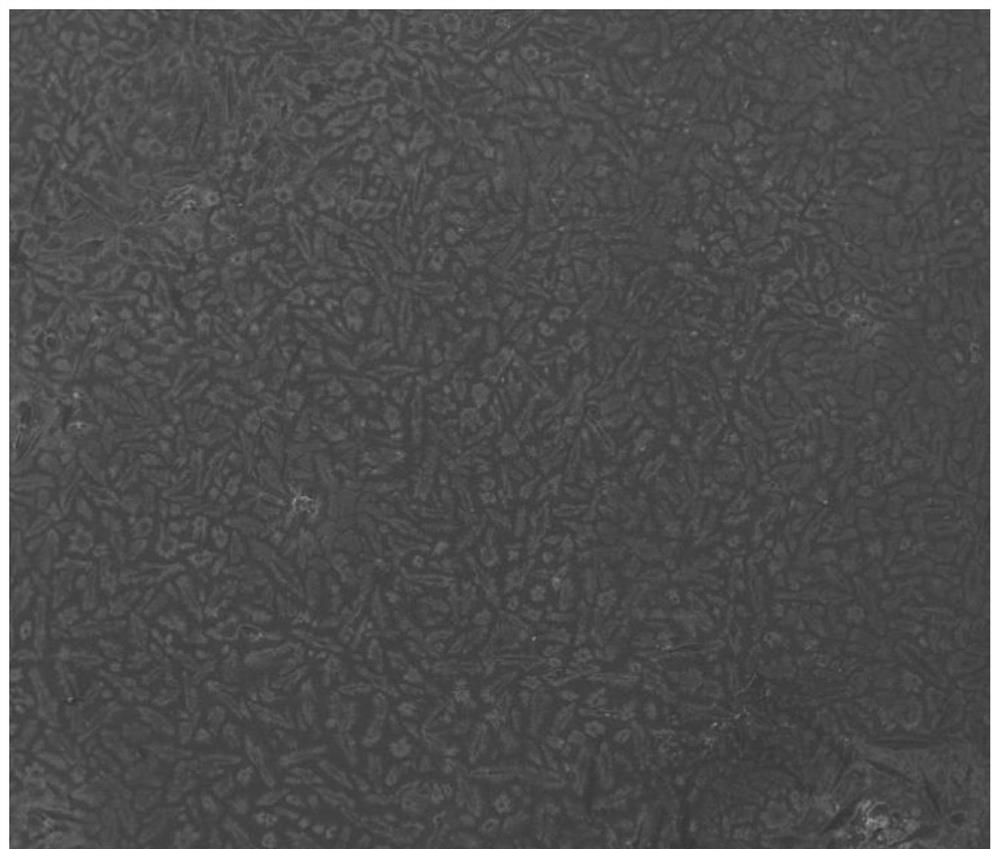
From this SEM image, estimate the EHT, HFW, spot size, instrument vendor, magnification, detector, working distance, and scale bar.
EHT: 15 kV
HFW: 2980 µm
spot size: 3.9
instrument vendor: FEI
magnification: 0.1 K X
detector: ETD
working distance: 6.3 mm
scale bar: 500000 nm